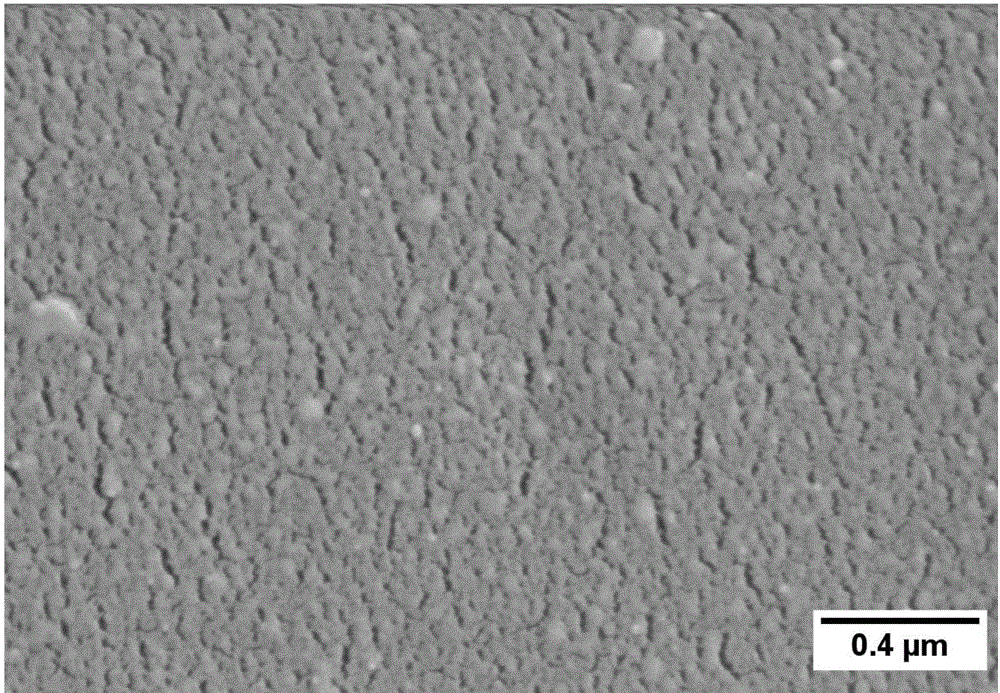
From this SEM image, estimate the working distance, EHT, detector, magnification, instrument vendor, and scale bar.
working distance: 10.6 mm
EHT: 3 kV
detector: SE(M)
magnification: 50 K X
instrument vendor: Hitachi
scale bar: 1000 nm